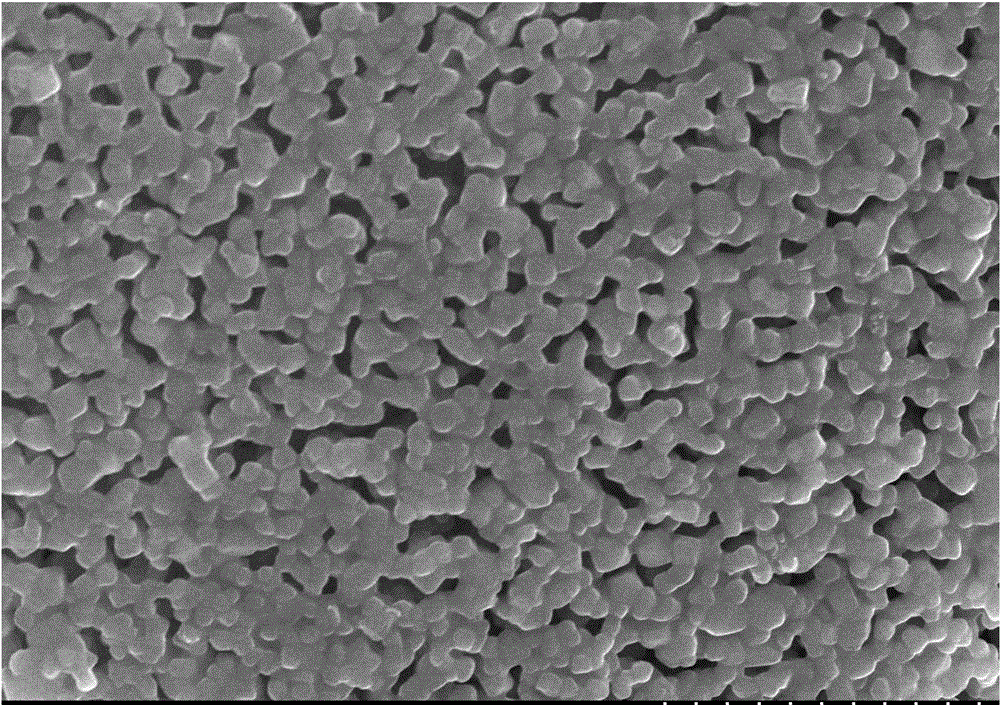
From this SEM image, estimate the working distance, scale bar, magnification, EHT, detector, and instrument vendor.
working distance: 8.5 mm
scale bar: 2000 nm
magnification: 20 K X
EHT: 10 kV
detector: SE(M)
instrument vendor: Hitachi